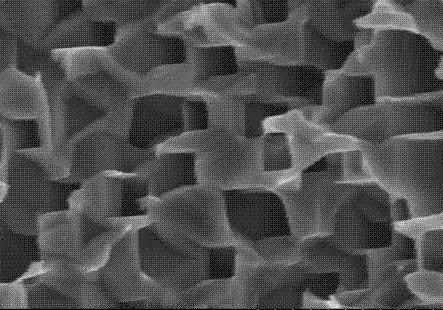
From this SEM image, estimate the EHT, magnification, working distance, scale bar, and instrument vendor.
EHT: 3 kV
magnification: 50 K X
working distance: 5.9 mm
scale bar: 1000 nm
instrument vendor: Hitachi